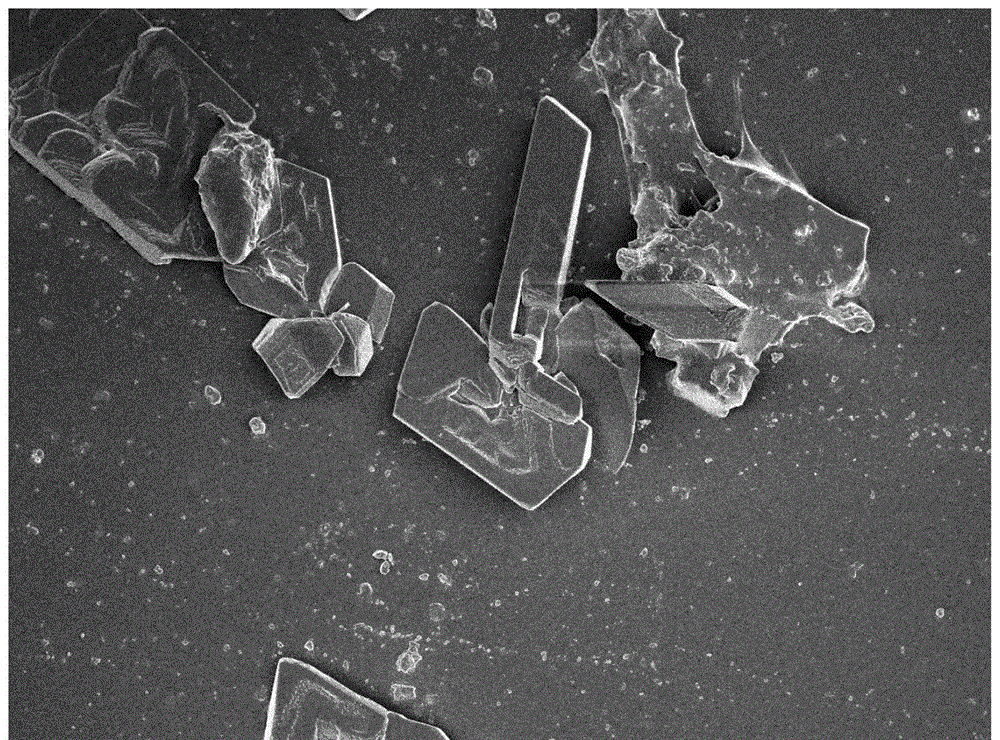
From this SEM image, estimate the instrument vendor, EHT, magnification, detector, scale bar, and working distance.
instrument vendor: JEOL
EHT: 3 kV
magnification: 1 K X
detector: SEI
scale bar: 10000 nm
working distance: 7.6 mm